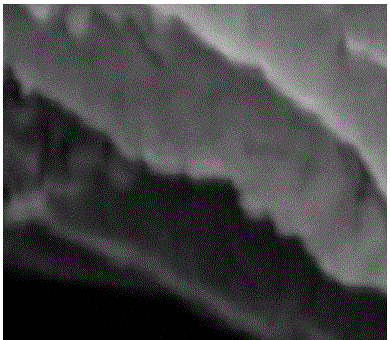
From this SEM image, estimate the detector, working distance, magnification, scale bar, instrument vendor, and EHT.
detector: SE(U)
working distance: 10.1 mm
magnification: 300 K X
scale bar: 100 nm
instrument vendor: Hitachi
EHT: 15 kV